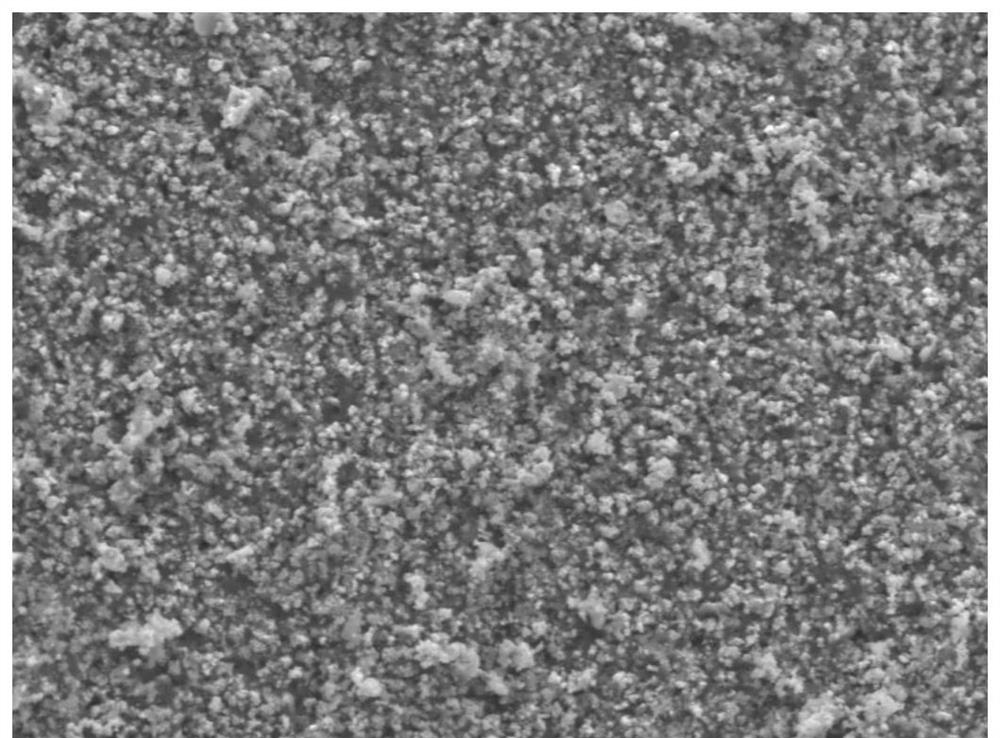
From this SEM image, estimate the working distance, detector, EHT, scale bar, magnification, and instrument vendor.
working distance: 8.1 mm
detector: LED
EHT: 3 kV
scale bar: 1000 nm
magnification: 10 K X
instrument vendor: JEOL